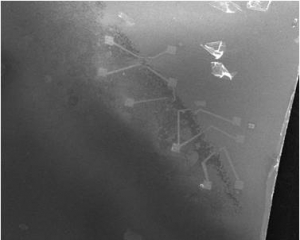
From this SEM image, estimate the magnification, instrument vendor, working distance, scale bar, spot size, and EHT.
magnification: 0.08 K X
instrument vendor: FEI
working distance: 10.1 mm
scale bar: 1000000 nm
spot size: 3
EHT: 20 kV